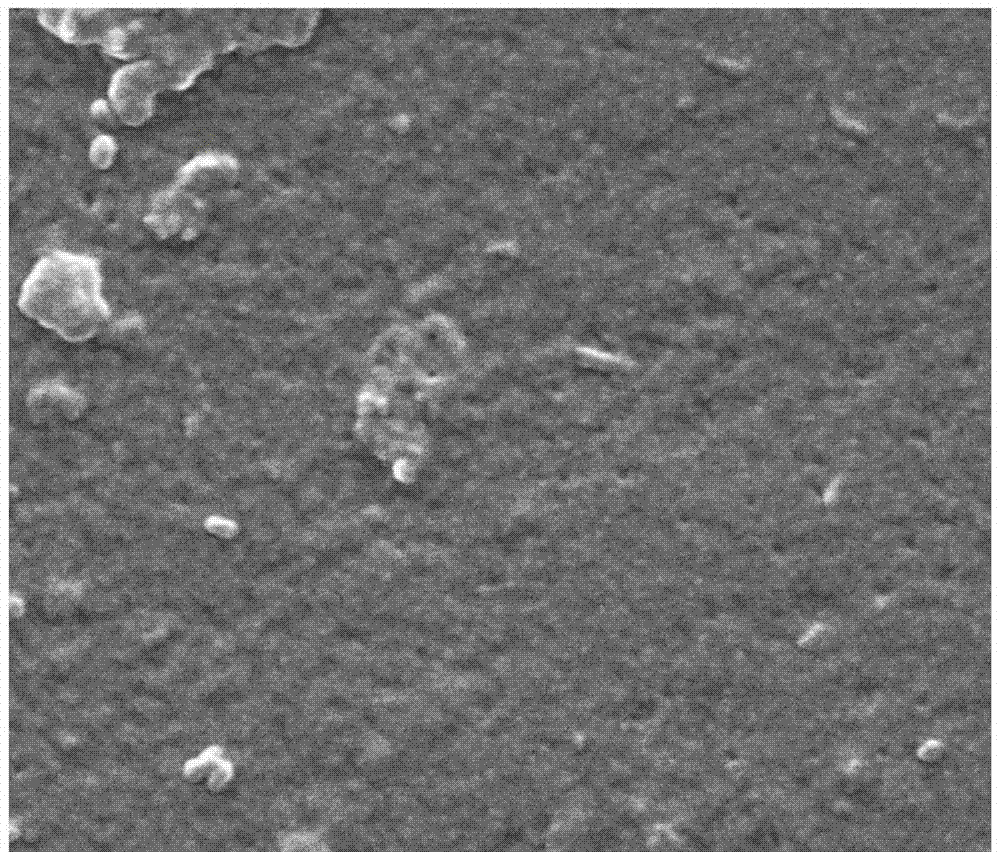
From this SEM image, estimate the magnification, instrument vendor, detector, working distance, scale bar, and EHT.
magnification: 100 K X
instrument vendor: FEI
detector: SE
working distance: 11 mm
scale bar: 1000 nm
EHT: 30 kV